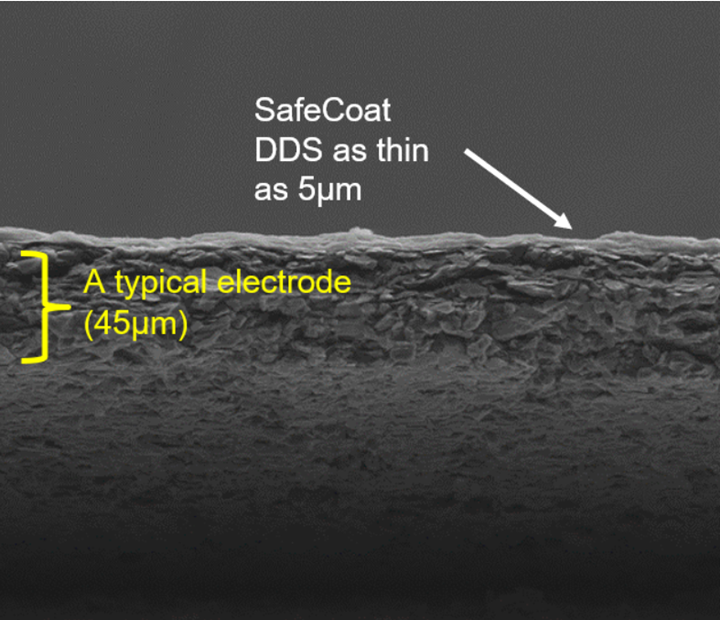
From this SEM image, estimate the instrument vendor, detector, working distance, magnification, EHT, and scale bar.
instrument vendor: FEI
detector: LVD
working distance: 4.5 mm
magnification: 0.718 K X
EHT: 15 kV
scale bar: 100000 nm